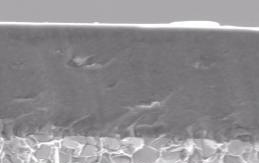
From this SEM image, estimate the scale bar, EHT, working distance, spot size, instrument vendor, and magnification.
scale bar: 2000 nm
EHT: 5 kV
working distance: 10.1 mm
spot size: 3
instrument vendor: FEI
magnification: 15 K X